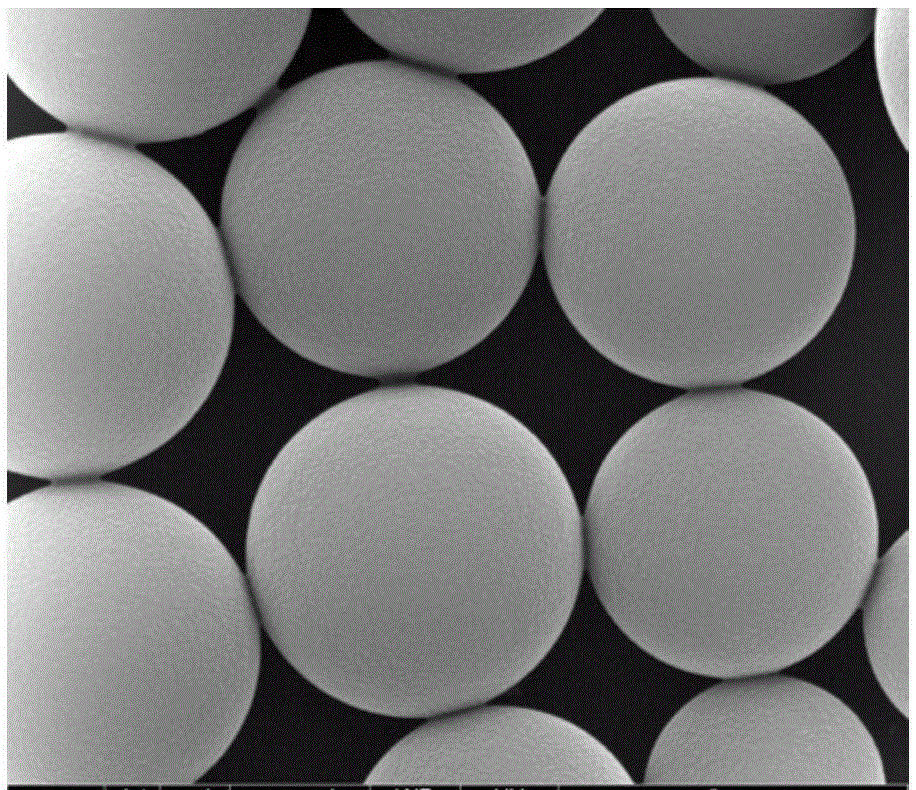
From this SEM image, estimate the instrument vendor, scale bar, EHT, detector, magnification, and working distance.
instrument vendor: FEI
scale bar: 2000 nm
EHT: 10 kV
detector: ETD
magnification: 50 K X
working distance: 9.1 mm